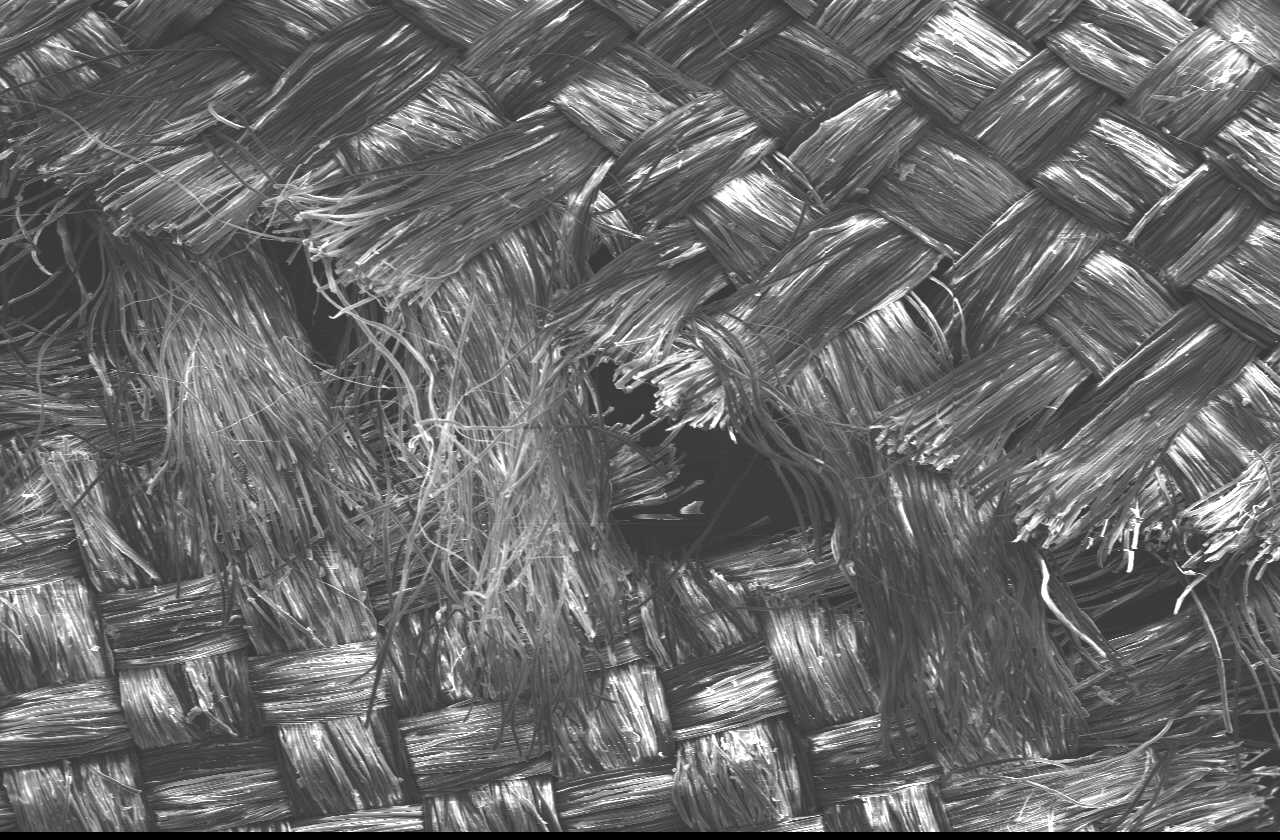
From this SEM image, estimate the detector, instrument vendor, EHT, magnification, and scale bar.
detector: SEI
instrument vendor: JEOL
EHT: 15 kV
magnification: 0.03 K X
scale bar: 500000 nm